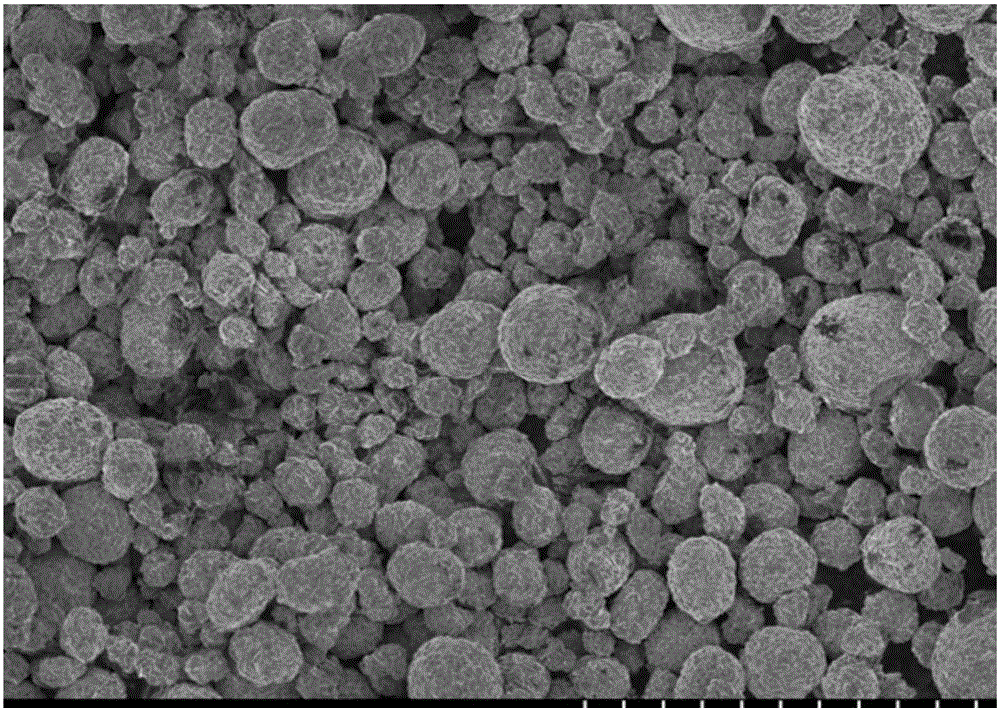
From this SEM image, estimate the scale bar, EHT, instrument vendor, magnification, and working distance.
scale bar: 50000 nm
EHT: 3 kV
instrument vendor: Hitachi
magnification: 1 K X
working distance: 8.3 mm